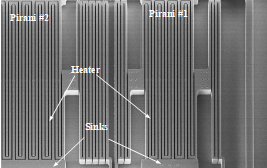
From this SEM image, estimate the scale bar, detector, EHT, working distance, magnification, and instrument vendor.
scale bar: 200000 nm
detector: SE(M)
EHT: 5 kV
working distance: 8.8 mm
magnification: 0.25 K X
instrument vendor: Hitachi